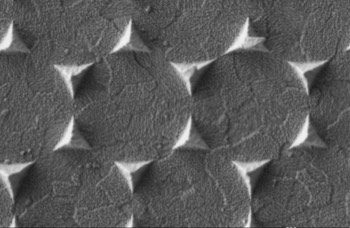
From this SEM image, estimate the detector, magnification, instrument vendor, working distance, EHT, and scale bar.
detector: SE2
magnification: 40 K X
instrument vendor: Zeiss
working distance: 3.8 mm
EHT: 1 kV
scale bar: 200 nm